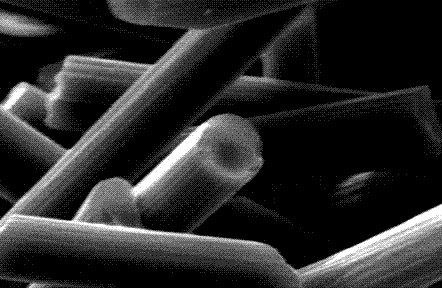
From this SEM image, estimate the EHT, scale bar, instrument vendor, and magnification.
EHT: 20 kV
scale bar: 5000 nm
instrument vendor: JEOL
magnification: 3 K X